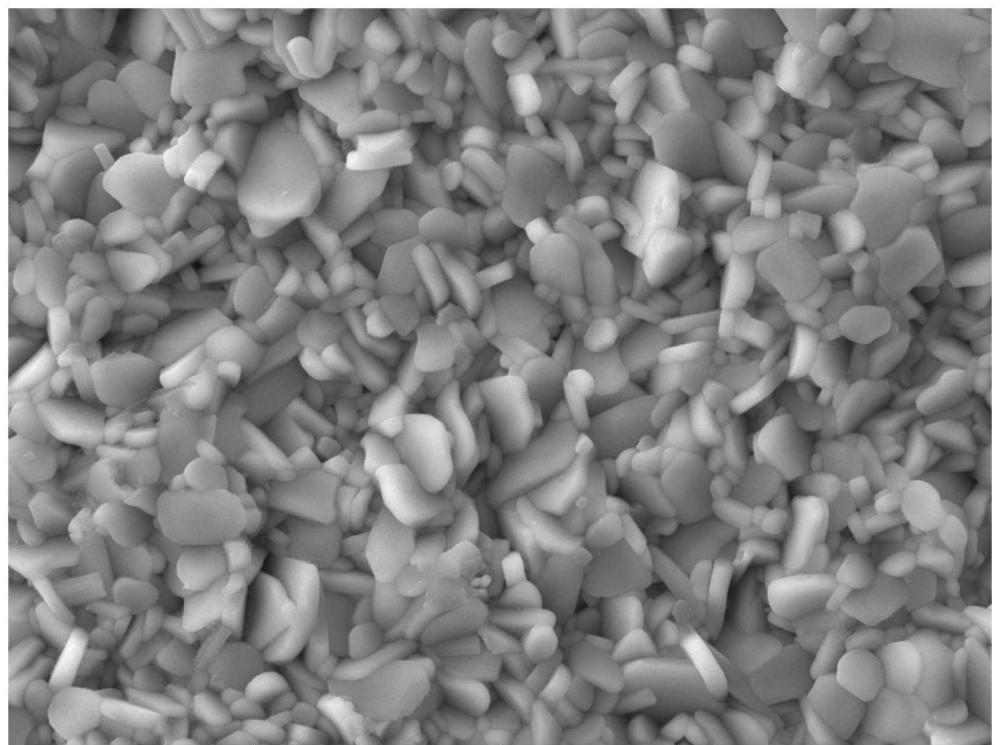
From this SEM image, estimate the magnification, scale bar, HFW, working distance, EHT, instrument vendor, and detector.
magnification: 10 K X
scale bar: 5000 nm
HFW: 27.7 µm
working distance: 15.58 mm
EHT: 20 kV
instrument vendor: Tescan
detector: SE